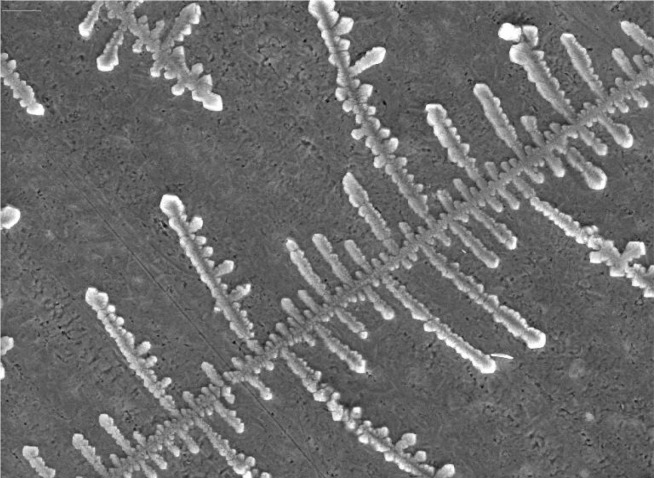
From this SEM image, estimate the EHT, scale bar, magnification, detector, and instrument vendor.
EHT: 30 kV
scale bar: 100000 nm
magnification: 0.57 K X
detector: SE Detector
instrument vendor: Tescan